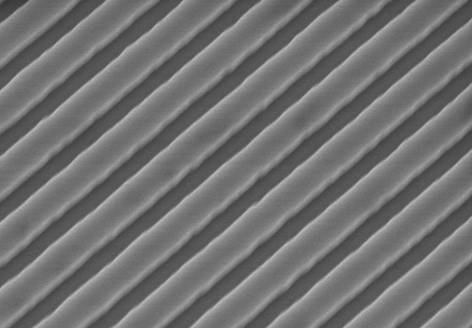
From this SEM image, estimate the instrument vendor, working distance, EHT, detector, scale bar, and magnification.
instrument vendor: Hitachi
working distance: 8.4 mm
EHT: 3 kV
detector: SE(U)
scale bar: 1000 nm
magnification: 50 K X